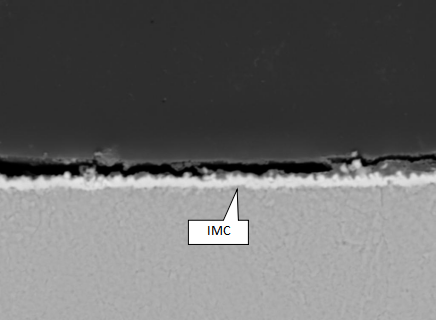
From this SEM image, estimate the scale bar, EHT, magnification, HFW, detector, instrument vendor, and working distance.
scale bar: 10000 nm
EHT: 20 kV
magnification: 5.5 K X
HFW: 50.3 µm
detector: BSE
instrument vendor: Tescan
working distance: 15.13 mm